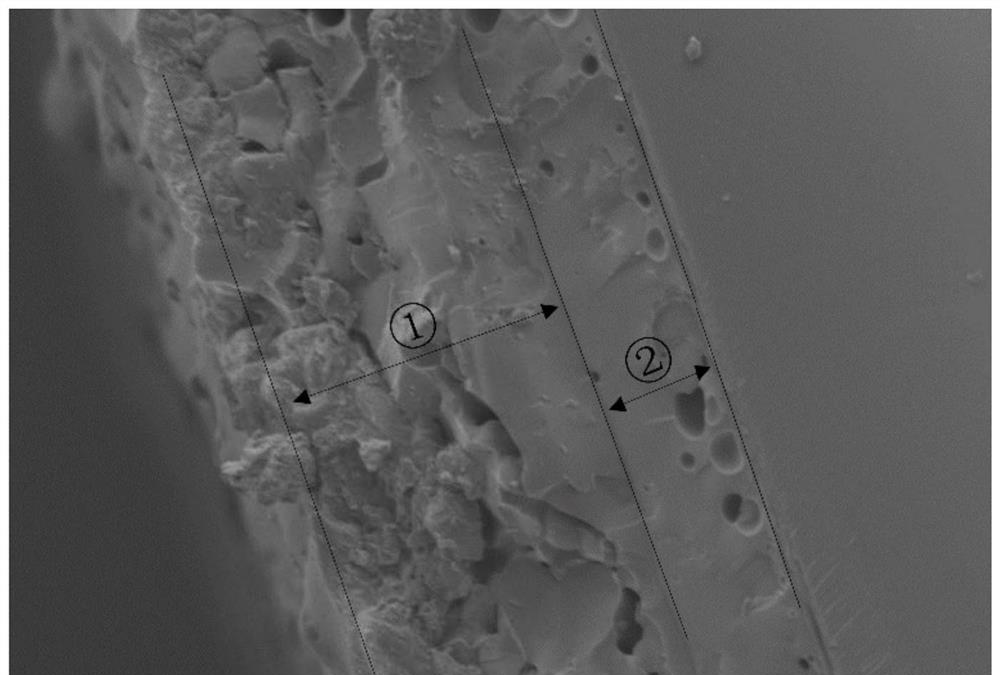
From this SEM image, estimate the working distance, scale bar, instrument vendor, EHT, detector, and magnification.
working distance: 16.3 mm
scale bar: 50000 nm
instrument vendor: Hitachi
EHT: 5 kV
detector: SE(M)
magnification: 1 K X